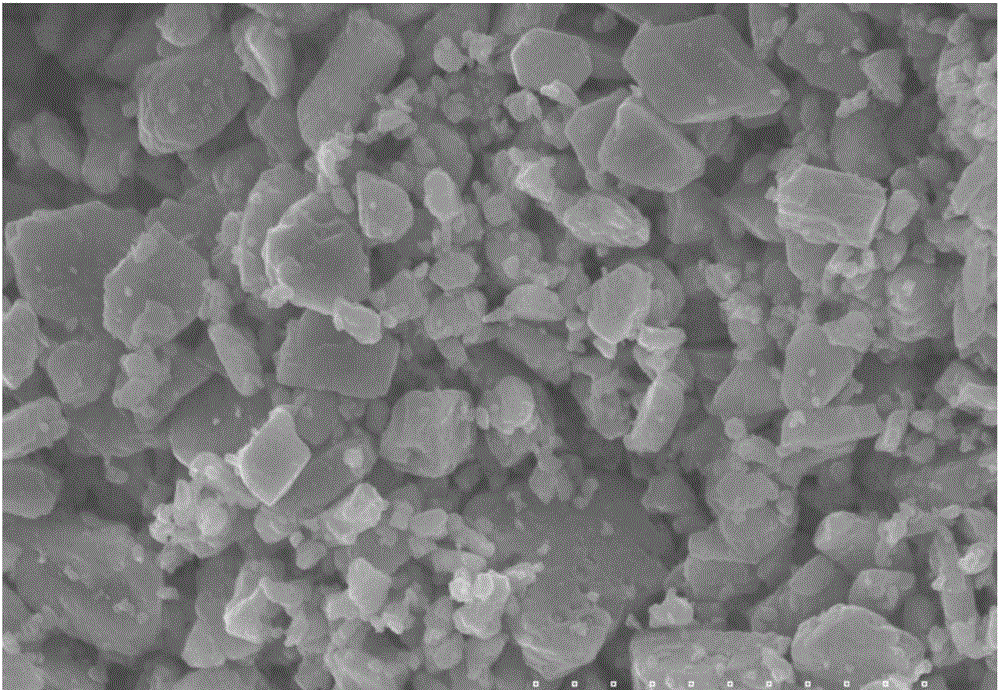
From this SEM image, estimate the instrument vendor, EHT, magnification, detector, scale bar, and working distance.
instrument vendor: Hitachi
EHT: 25 kV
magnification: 1 K X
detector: SE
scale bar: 30000 nm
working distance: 13.8 mm